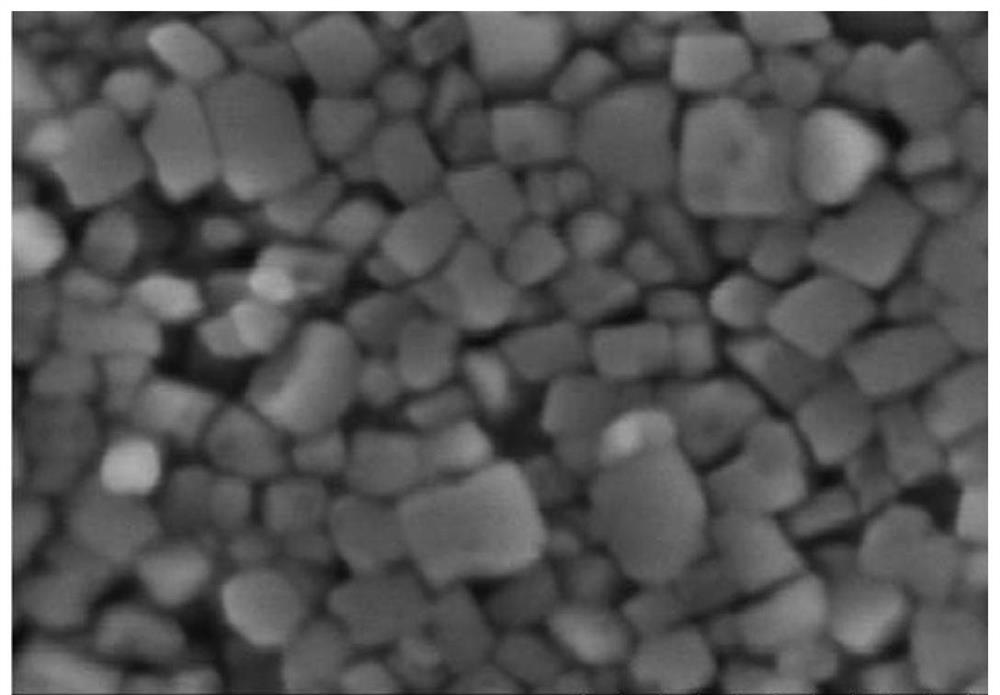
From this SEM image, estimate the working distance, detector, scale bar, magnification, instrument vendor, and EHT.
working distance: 8.4 mm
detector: SE(M)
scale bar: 200 nm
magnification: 250 K X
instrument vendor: Hitachi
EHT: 3 kV